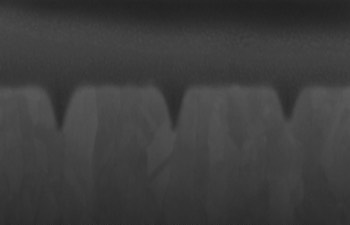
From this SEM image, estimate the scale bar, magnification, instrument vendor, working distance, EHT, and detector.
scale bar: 100 nm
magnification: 100 K X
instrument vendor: Zeiss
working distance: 6.4 mm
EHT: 5 kV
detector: InLens